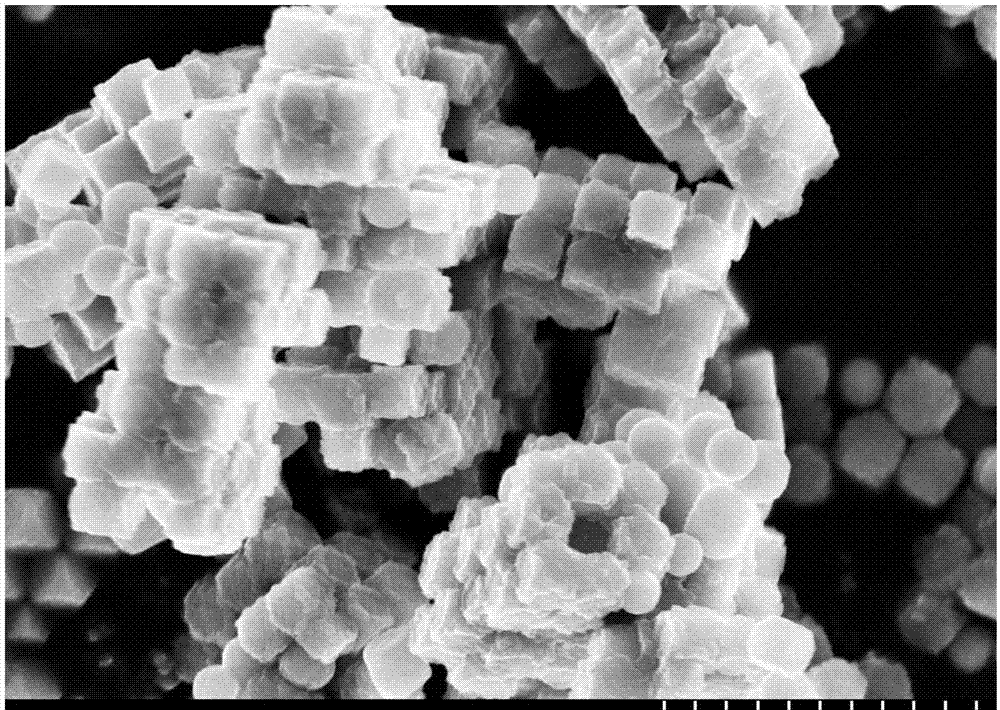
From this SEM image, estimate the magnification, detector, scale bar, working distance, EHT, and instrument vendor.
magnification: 20 K X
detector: SE(M)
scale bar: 2000 nm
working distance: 7.8 mm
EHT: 5 kV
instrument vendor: Hitachi